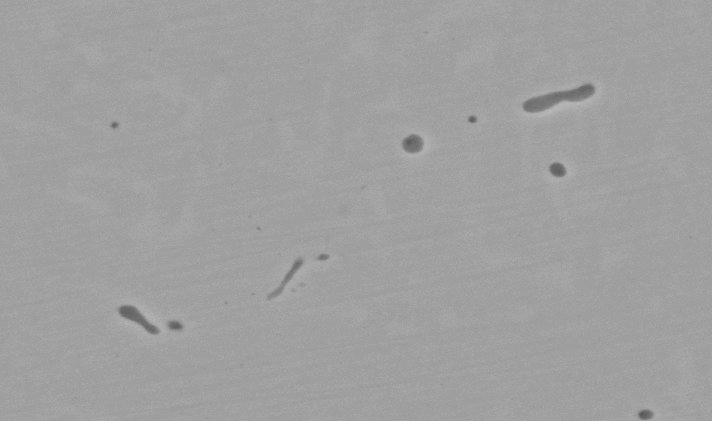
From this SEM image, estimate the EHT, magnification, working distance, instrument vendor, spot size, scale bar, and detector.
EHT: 20 kV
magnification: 8 K X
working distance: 10.1 mm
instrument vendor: FEI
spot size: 4.4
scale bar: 5000 nm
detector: BSE